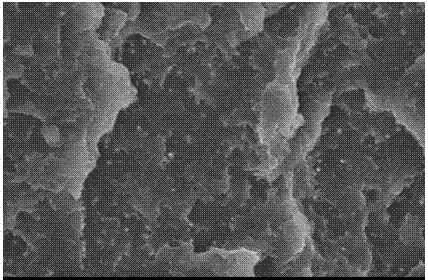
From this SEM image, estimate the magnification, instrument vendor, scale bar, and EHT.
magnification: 2 K X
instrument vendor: JEOL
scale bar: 10000 nm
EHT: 20 kV